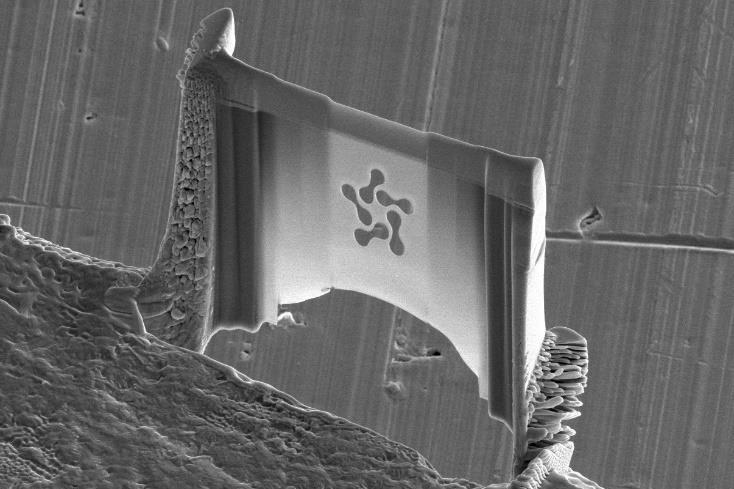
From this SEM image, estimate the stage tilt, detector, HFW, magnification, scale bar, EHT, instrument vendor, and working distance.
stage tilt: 52°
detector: ETD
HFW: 25.9 µm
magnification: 8 K X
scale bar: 5000 nm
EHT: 5 kV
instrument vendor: FEI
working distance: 3.9 mm